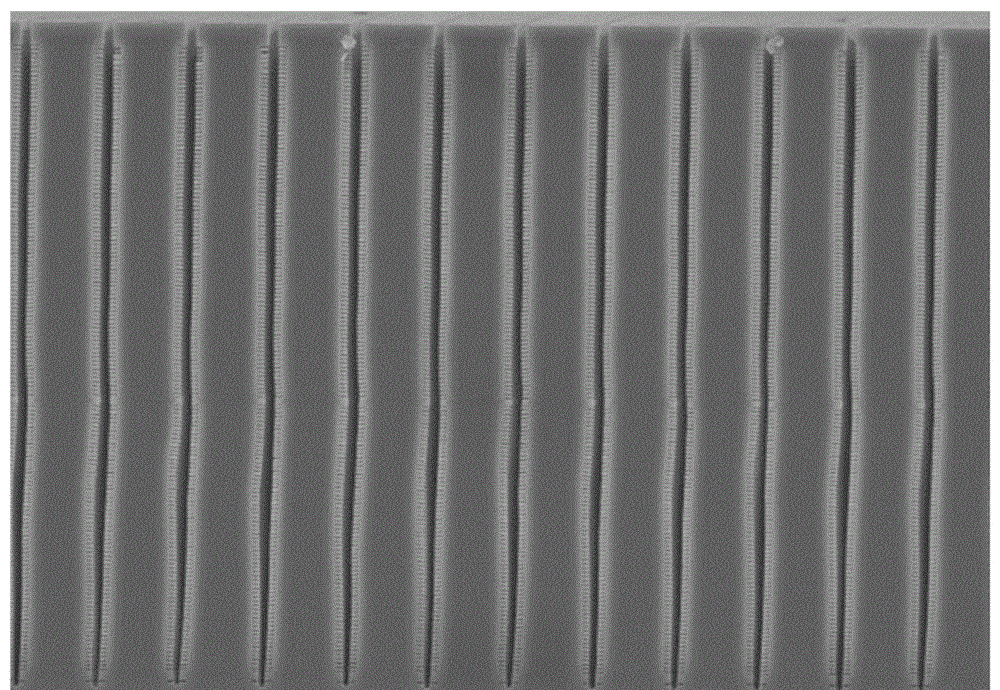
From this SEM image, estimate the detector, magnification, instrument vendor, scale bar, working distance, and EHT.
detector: SE(M)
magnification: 7 K X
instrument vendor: Hitachi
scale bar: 5000 nm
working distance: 8.1 mm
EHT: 5 kV